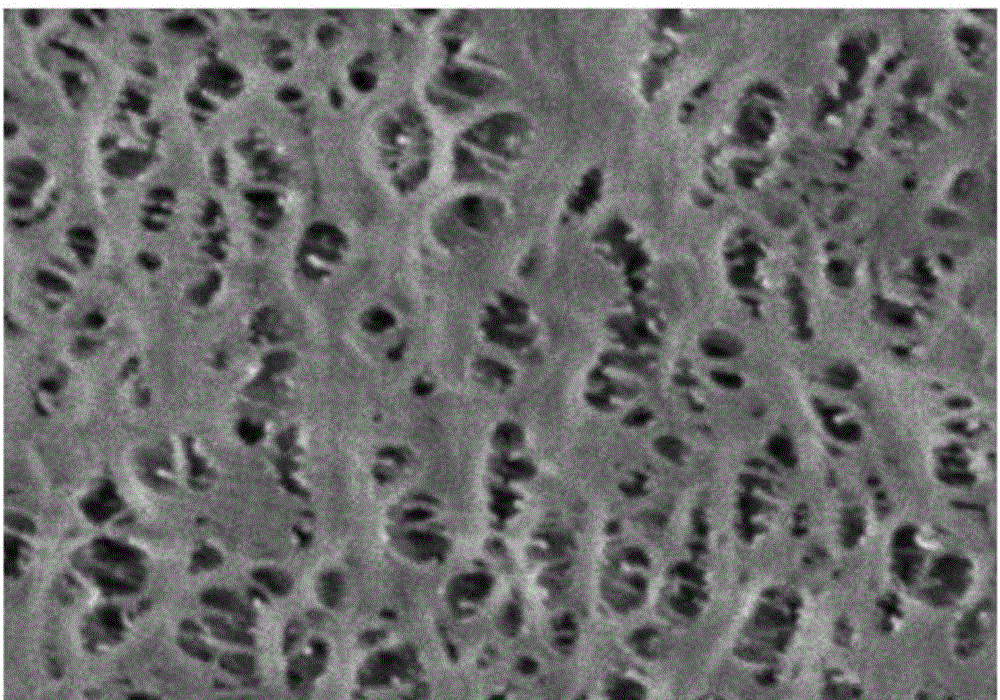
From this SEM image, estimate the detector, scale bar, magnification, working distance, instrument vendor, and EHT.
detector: SE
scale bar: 2000 nm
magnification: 20 K X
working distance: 5.9 mm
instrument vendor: Hitachi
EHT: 3 kV